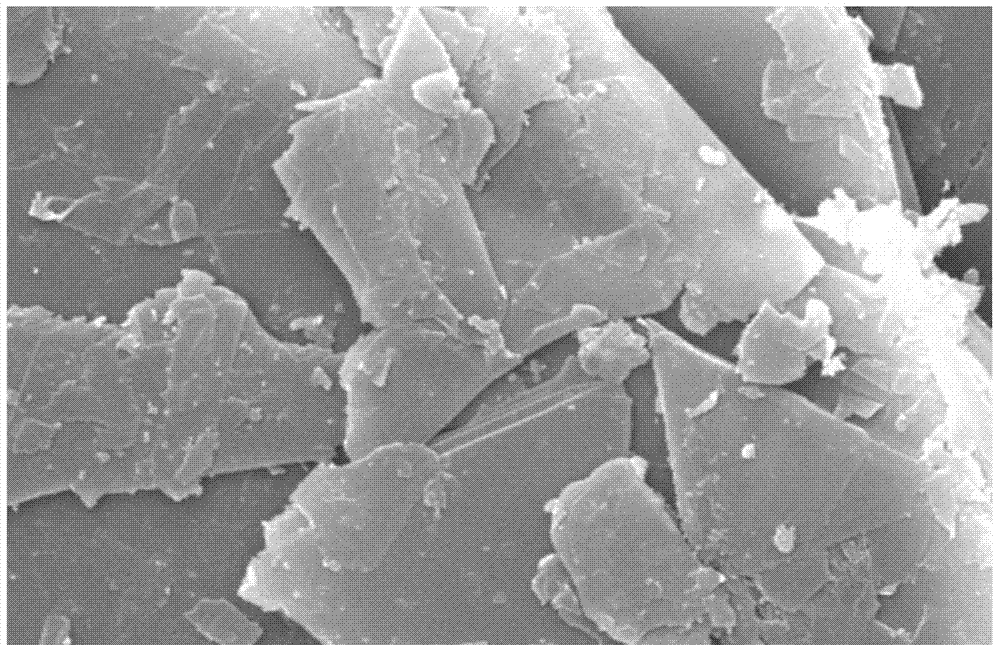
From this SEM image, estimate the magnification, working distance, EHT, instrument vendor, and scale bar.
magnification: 8 K X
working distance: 9.8 mm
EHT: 20 kV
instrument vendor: FEI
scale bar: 5000 nm